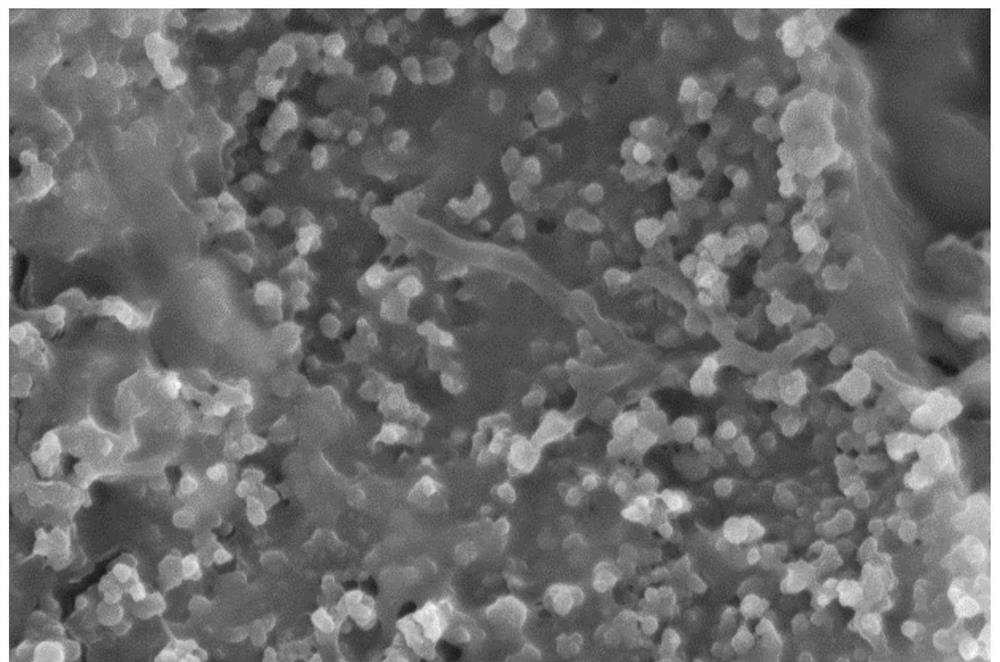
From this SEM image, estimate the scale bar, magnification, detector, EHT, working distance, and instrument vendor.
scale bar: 10000 nm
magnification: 5 K X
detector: SE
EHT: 15 kV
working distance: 13.2 mm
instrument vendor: Hitachi